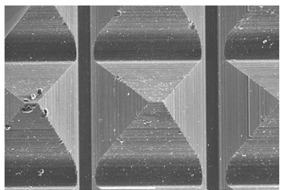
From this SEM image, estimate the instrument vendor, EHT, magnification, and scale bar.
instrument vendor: JEOL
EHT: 15 kV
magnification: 0.25 K X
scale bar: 100000 nm